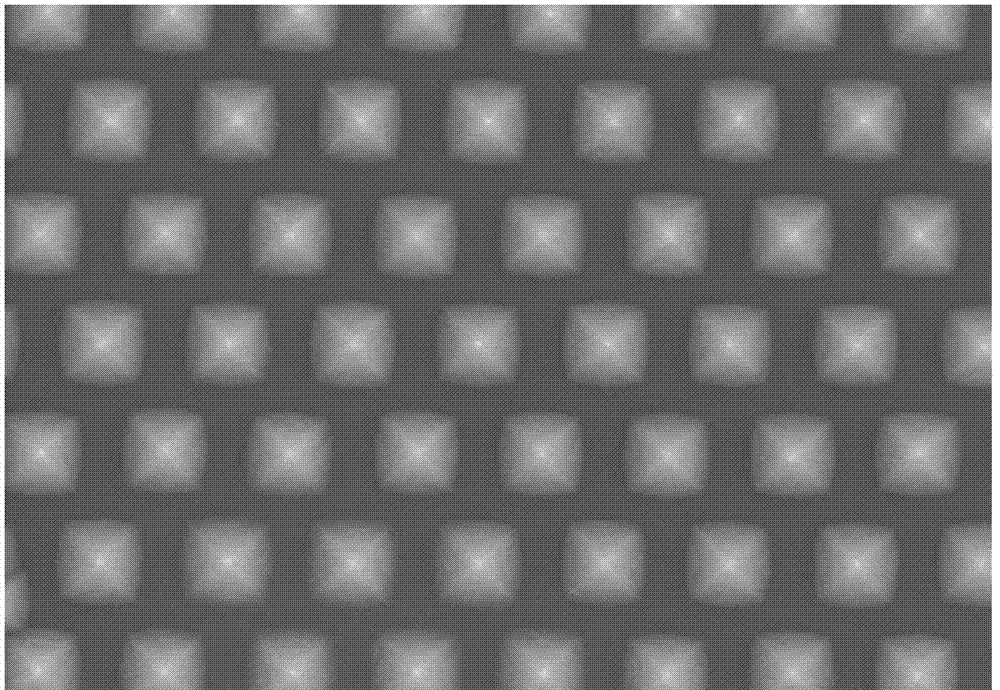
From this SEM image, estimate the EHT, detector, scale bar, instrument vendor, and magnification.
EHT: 10 kV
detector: SE(M)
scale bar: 5000 nm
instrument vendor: Hitachi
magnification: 7 K X